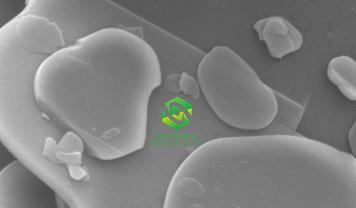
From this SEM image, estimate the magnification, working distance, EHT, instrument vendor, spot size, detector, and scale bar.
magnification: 10 K X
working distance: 6.7 mm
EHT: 15 kV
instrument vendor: FEI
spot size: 4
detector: SE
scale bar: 5000 nm